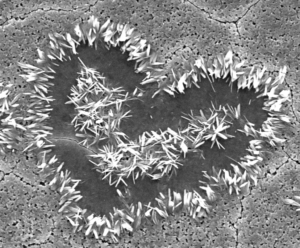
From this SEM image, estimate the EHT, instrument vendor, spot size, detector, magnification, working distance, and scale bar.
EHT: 10 kV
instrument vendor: FEI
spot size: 2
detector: ETD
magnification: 5.489 K X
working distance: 9.9 mm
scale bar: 5000 nm